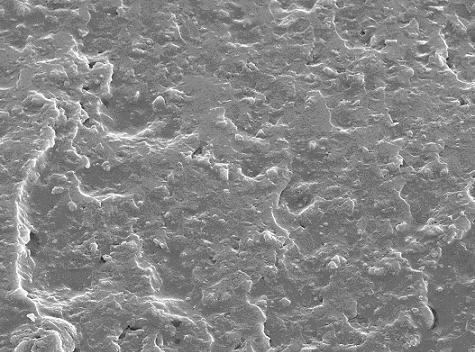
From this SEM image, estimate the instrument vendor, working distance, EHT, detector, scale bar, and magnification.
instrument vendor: JEOL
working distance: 35.2 mm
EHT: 10 kV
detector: SEI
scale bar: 10000 nm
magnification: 2 K X